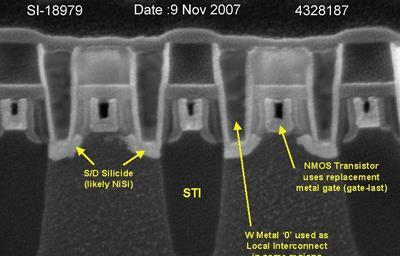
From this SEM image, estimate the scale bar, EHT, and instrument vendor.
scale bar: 100 nm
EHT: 5 kV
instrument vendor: Zeiss